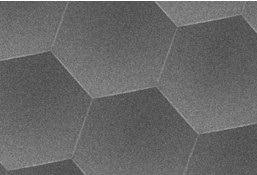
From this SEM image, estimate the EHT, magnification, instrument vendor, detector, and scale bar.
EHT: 3 kV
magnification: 0.5 K X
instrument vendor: Hitachi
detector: SE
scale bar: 100000 nm